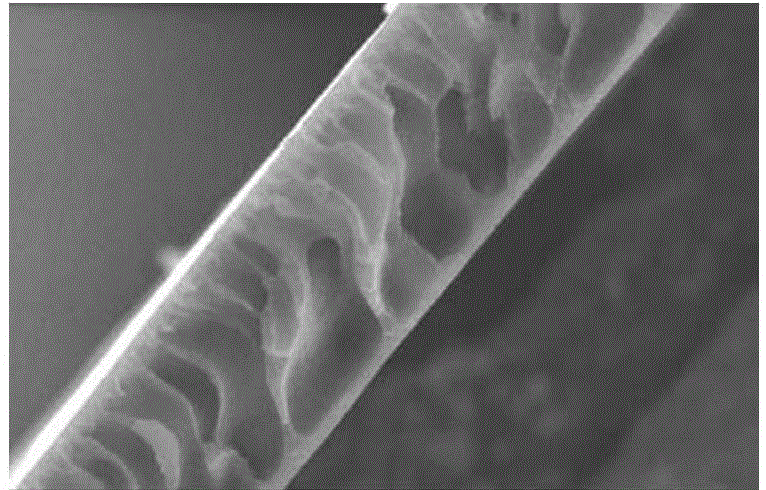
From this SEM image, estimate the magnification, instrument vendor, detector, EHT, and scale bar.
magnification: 0.3 K X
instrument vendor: JEOL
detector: SEI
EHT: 30 kV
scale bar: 50000 nm